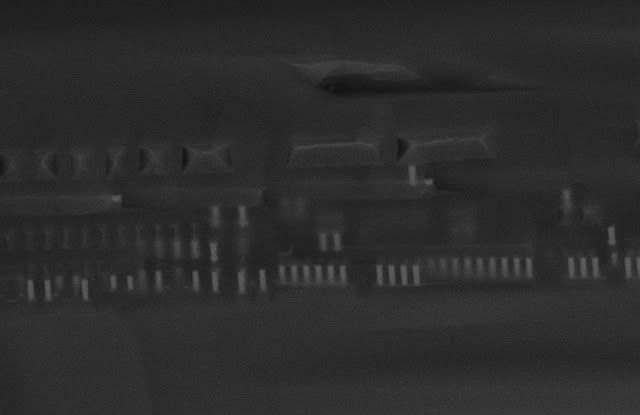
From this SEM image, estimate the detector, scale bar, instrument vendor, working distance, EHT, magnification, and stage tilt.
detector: InLens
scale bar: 2000 nm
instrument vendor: Zeiss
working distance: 4 mm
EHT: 15 kV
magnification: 11.47 K X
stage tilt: -15°